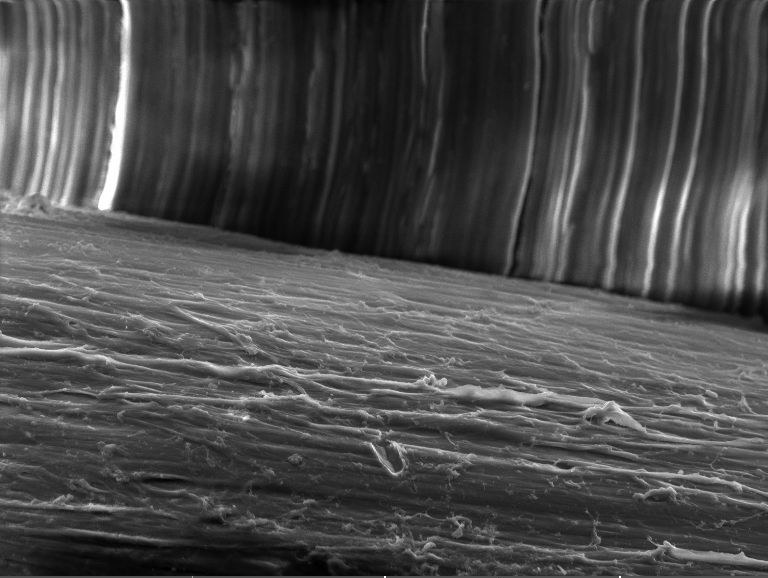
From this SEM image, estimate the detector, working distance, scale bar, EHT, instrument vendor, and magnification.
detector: SE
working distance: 8.67 mm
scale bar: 20000 nm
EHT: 10 kV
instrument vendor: Tescan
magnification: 2.92 K X